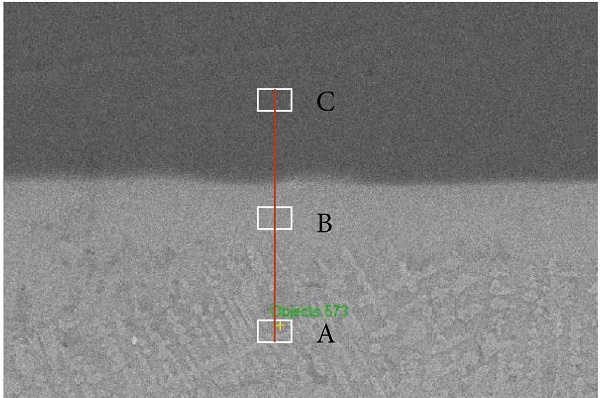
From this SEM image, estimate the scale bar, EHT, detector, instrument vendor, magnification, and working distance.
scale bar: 30000 nm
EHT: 20 kV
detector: SE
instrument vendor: Zeiss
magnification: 1 K X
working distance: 8.8 mm